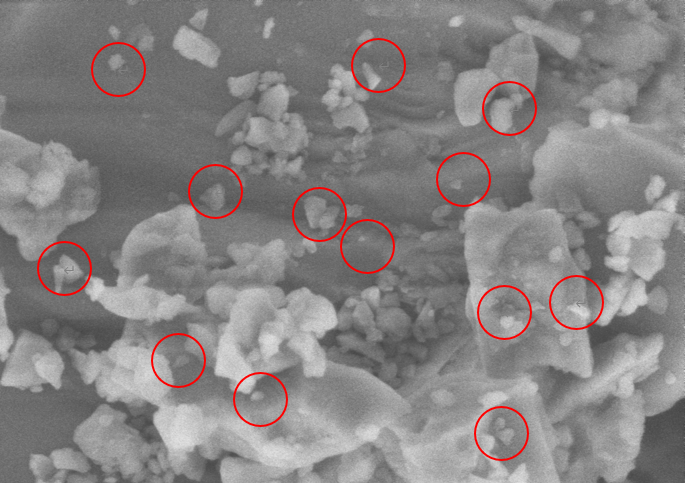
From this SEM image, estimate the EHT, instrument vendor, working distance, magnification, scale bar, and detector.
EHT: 3 kV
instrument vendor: Zeiss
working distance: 6 mm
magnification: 50 K X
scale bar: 200 nm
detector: SE2